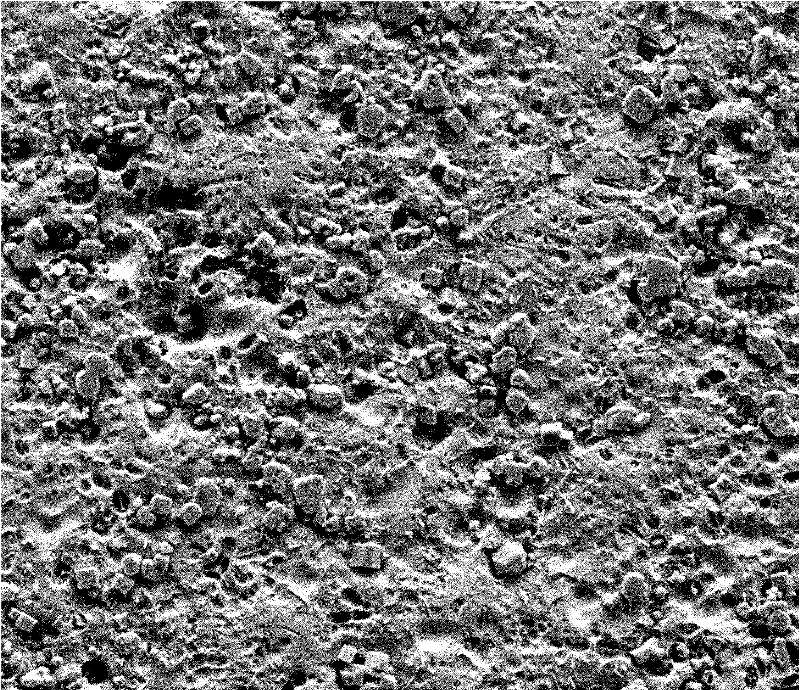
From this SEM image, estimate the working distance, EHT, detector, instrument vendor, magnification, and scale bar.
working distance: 12.1 mm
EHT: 20 kV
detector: SE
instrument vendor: FEI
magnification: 1 K X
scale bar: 100000 nm